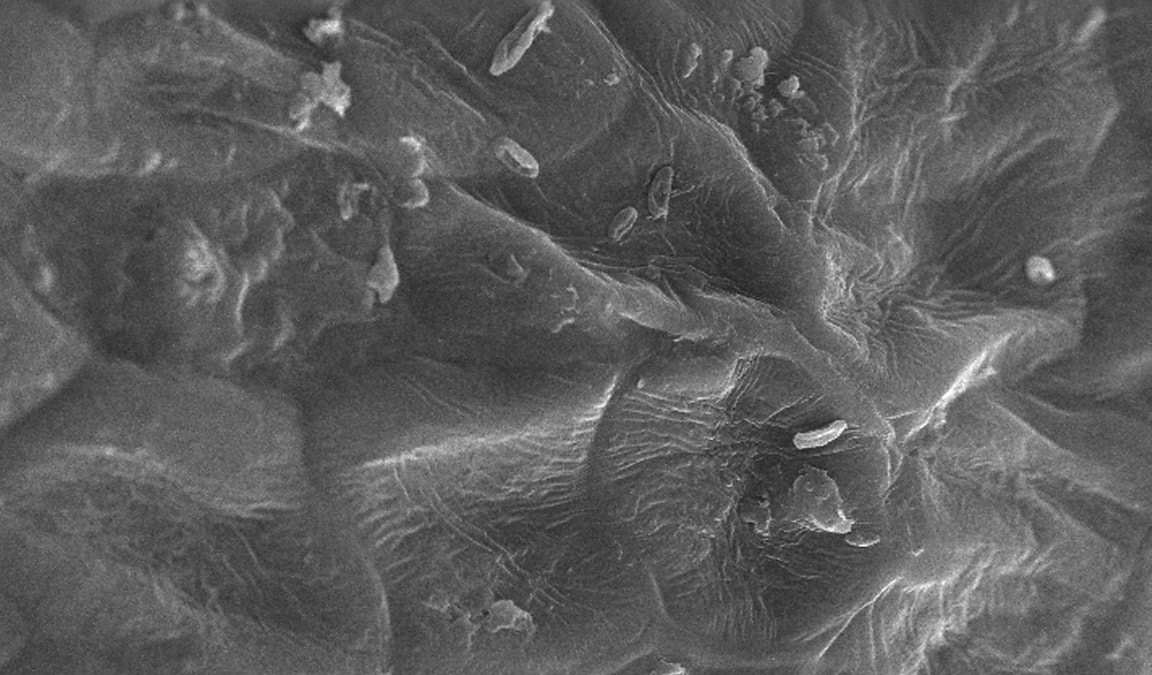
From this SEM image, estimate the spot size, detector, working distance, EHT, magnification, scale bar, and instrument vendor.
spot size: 3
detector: SE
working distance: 3.2 mm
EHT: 30 kV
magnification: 0.943 K X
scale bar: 20000 nm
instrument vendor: FEI